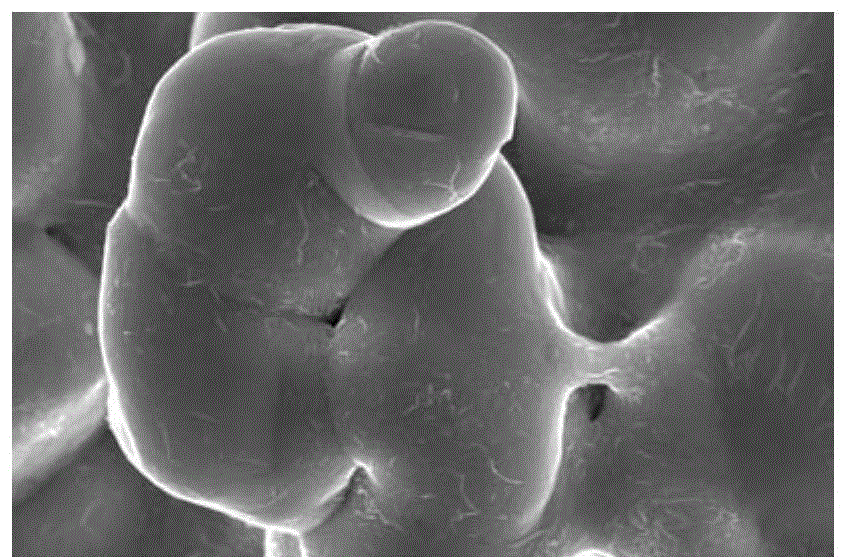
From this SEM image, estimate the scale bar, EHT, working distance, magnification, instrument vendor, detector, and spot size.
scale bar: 4000 nm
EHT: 20 kV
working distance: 6.6 mm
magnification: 40 K X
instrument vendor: FEI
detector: TLD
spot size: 5